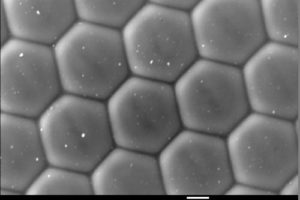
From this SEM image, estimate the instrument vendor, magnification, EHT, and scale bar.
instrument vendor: JEOL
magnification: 1 K X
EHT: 20 kV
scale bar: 10000 nm